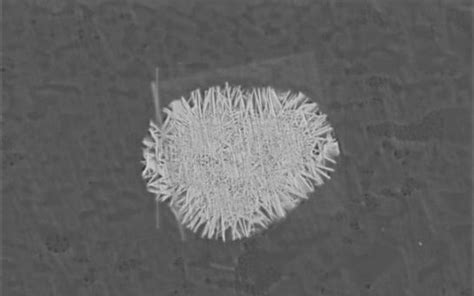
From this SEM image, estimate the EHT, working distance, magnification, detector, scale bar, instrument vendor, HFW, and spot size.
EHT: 2 kV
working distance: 5 mm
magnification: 20 K X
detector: T1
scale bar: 4000 nm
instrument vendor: Thermo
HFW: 10.4 µm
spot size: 6